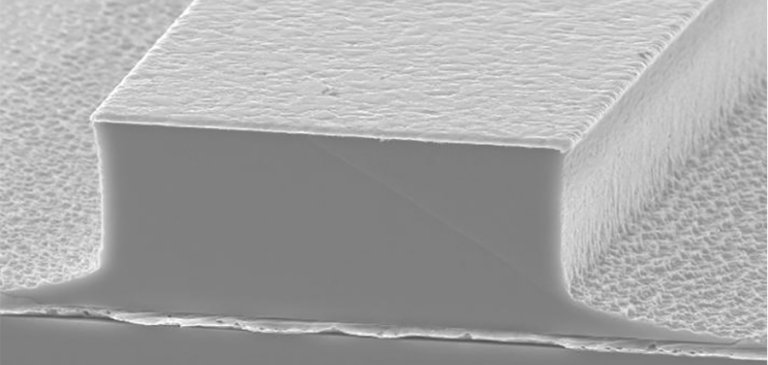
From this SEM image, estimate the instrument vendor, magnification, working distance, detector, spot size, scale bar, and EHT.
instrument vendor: FEI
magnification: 3.5 K X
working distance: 15.9 mm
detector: SE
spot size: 5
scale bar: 10000 nm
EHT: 15 kV